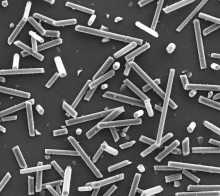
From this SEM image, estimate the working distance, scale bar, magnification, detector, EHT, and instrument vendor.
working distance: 10.5 mm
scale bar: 100000 nm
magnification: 0.5 K X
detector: ETD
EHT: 20 kV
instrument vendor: FEI